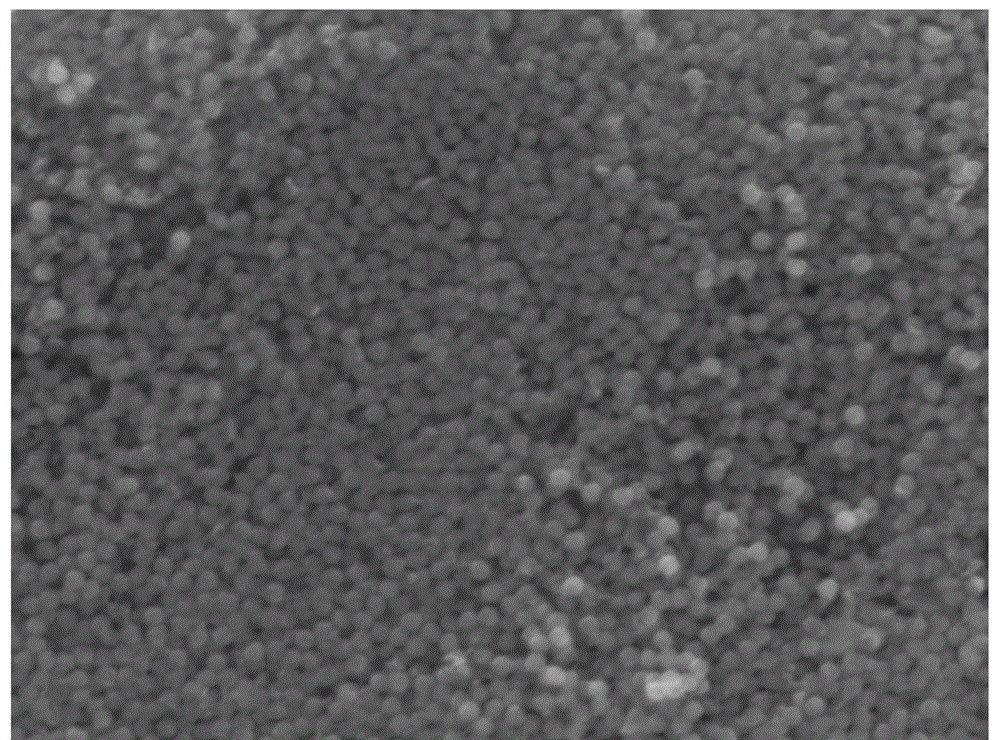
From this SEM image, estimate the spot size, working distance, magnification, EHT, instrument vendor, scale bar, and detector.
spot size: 5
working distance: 23.6 mm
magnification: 10 K X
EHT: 20 kV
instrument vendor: FEI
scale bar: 2000 nm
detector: SE